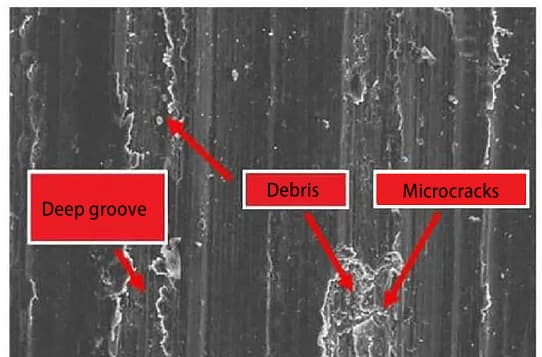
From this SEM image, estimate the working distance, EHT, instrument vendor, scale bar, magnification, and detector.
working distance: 25.5 mm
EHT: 20 kV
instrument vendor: JEOL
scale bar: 100000 nm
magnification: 0.18 K X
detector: SE